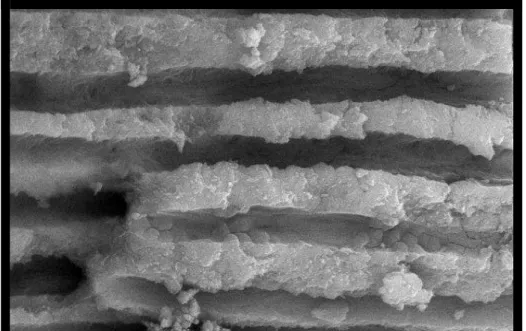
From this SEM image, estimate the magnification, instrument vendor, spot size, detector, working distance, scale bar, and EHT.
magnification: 10 K X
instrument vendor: FEI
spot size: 3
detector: BSE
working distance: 10.2 mm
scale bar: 5000 nm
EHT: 20 kV